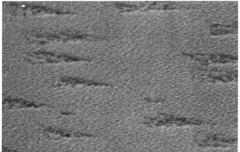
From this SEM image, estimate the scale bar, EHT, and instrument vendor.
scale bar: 1000 nm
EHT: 20 kV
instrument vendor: JEOL